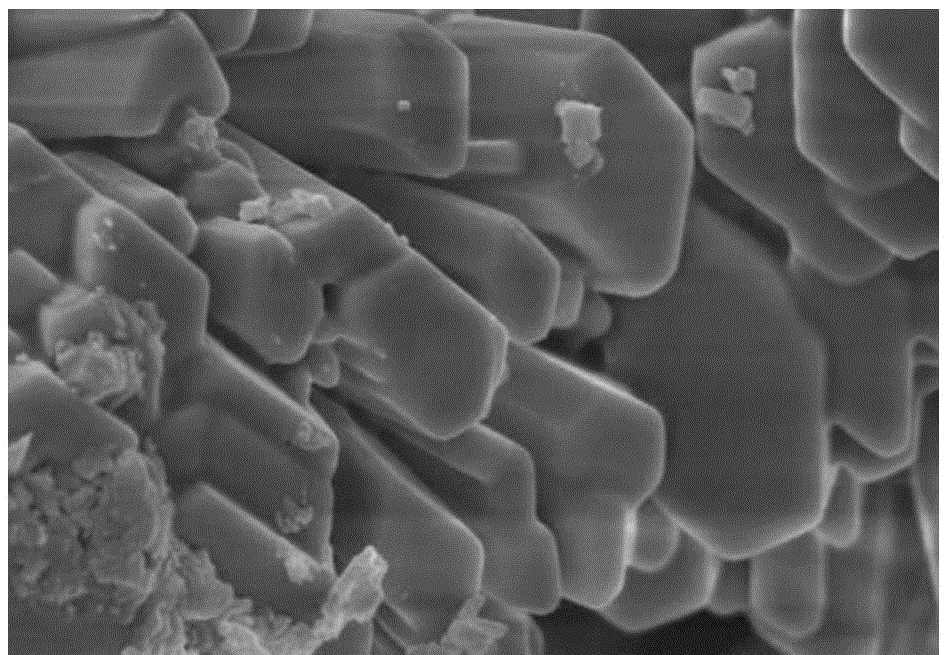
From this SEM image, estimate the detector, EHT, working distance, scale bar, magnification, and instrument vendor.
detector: SE(M,LA0)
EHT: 5 kV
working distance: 8 mm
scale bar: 2000 nm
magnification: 20 K X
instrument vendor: Hitachi